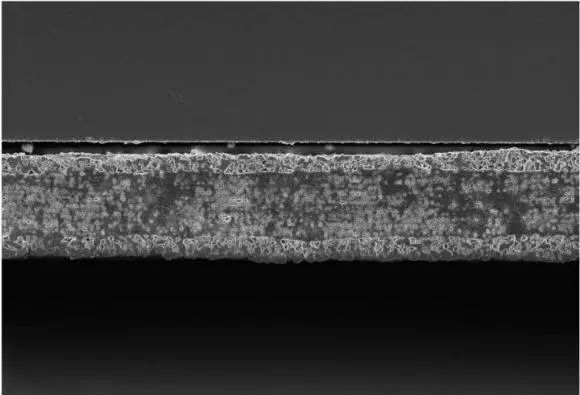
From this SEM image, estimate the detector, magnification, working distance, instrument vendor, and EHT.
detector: InLens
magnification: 2 K X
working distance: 2.3 mm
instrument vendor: Zeiss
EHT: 3 kV